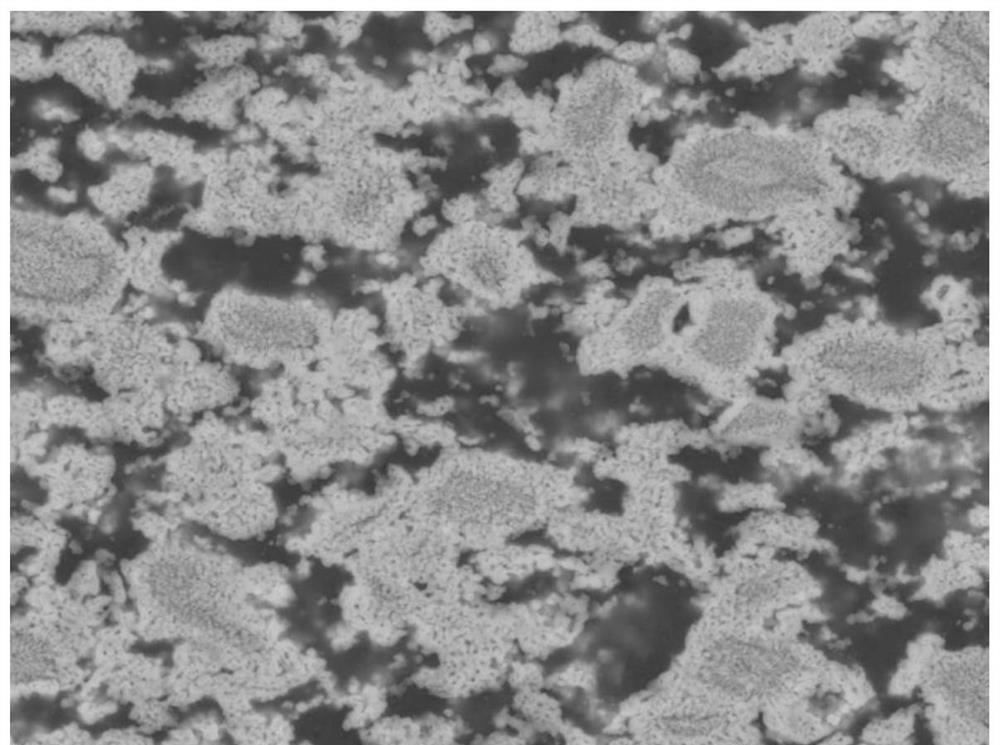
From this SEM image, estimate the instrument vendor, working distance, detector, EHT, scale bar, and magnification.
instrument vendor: JEOL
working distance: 10 mm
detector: COMPO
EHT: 20 kV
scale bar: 1000 nm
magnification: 3 K X